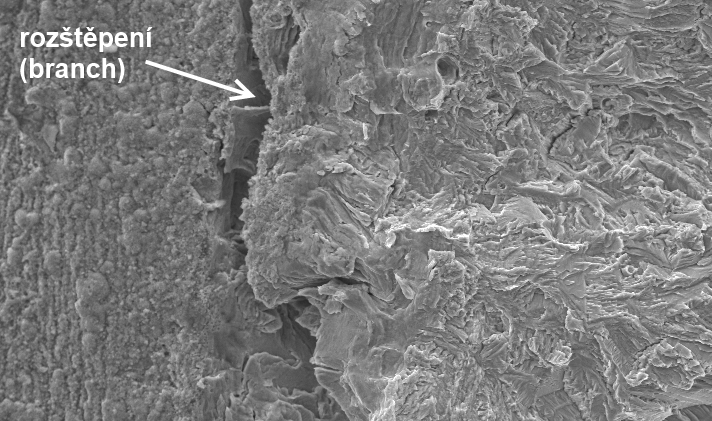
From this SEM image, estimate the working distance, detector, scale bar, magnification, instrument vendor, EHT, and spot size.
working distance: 10.1 mm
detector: SE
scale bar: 50000 nm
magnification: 0.5 K X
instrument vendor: FEI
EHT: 20 kV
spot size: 4